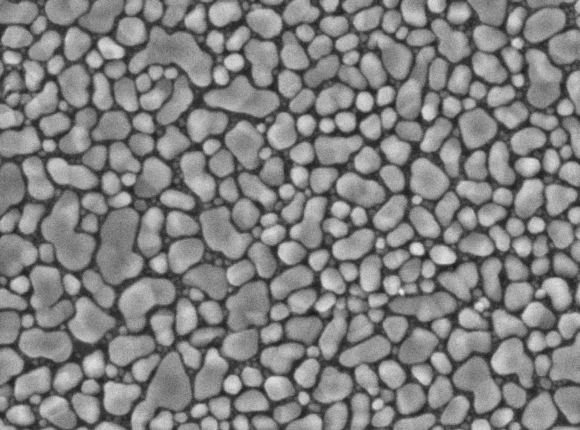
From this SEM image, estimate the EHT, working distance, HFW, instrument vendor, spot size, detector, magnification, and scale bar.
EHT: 20 kV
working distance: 5.02 mm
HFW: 3.51 µm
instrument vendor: other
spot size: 2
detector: SE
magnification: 100 K X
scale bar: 200 nm